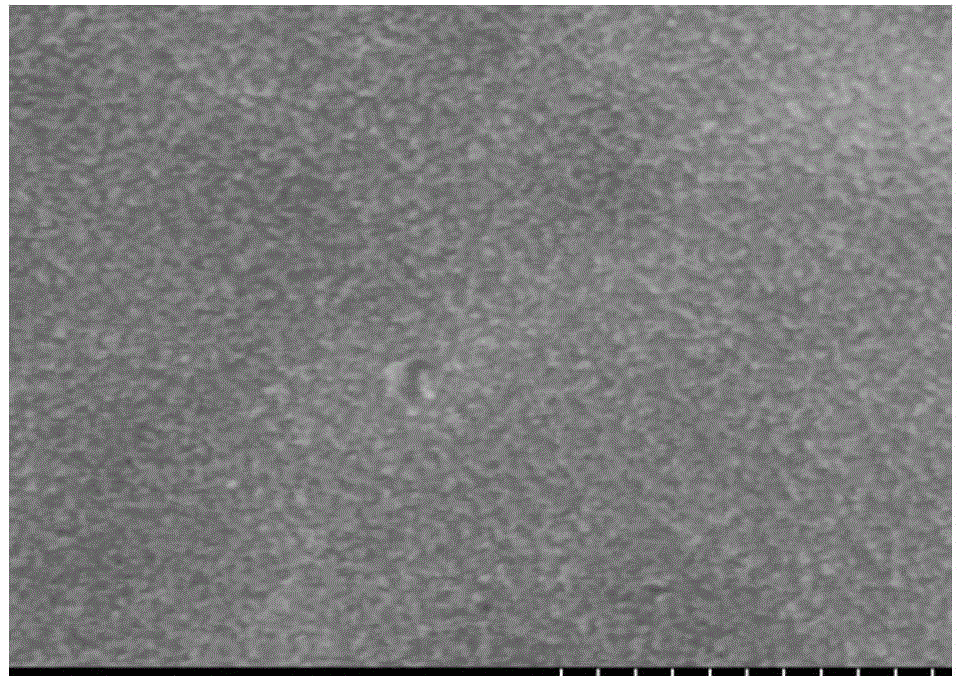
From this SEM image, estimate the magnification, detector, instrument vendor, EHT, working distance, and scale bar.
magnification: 100 K X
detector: SE(M,LA0)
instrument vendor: Hitachi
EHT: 5 kV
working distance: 10.9 mm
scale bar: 500 nm